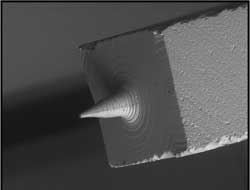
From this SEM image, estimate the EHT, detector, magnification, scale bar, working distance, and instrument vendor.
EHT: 25 kV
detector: BSE1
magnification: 0.025 K X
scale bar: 2000000 nm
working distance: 33.8 mm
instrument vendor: Hitachi